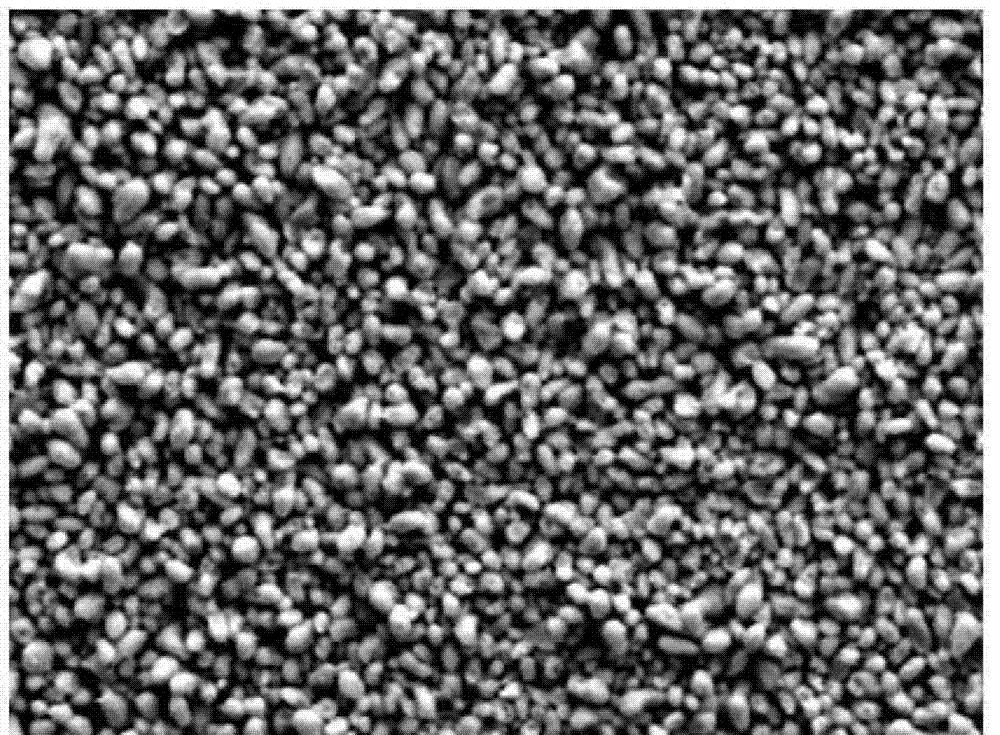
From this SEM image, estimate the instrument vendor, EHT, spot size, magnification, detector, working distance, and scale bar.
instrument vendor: FEI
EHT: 20 kV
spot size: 6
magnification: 2 K X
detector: SE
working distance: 23.6 mm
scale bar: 10000 nm